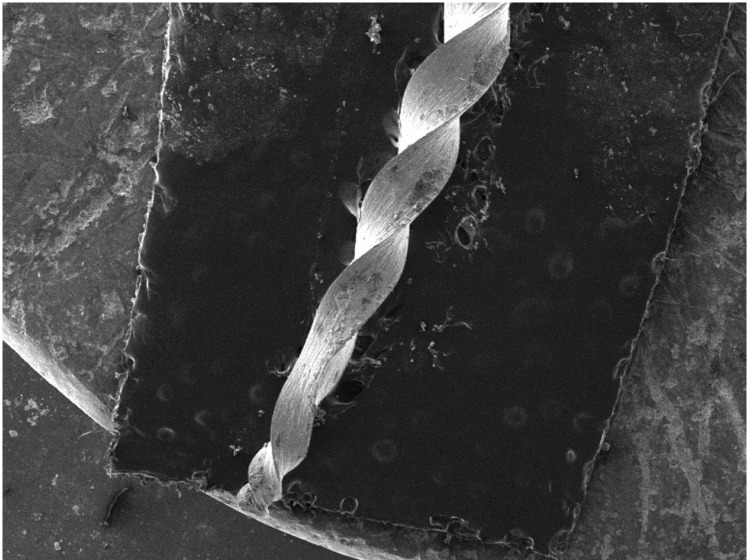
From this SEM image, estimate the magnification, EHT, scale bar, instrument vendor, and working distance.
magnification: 0.041 K X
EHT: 10 kV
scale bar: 1000000 nm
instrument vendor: Tescan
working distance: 15.51 mm